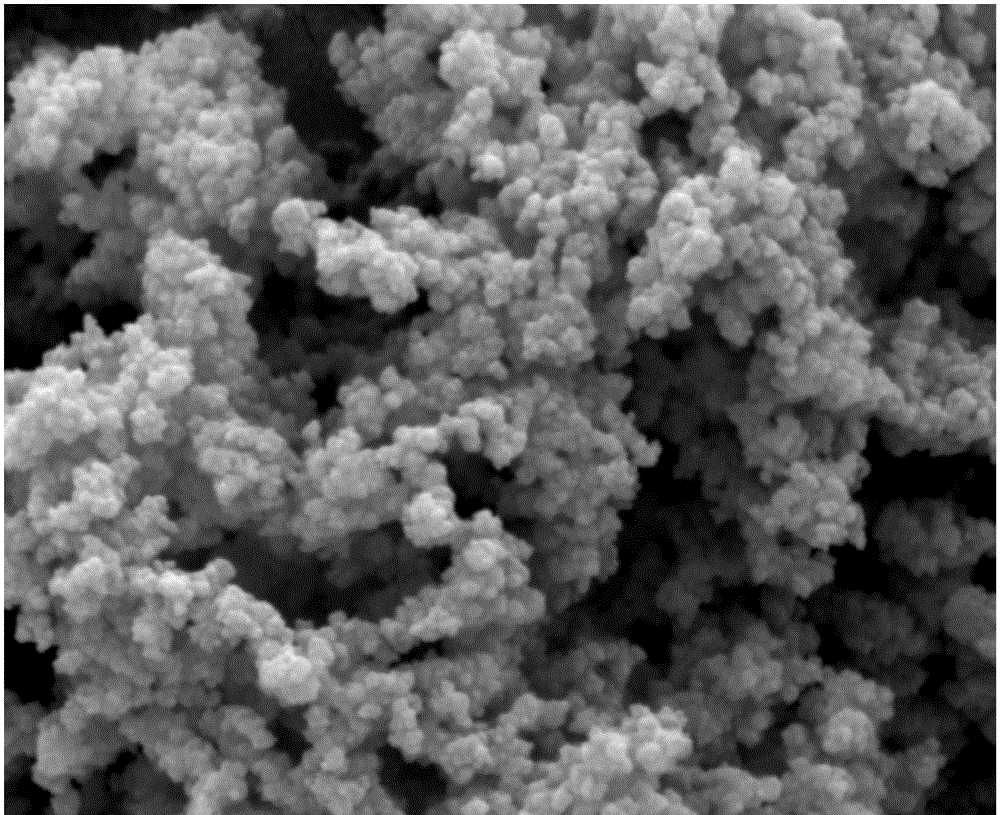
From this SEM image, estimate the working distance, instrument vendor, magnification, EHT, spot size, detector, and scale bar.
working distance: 9.8 mm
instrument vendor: FEI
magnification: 80 K X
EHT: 5 kV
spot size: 3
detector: ETD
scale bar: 1000 nm